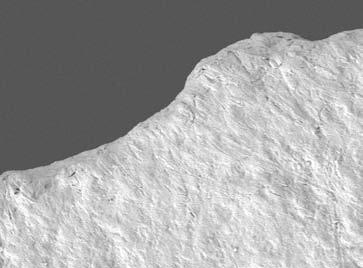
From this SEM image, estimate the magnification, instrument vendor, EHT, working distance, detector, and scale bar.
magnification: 1.5 K X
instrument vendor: JEOL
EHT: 5 kV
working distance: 9.6 mm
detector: LEI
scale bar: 10000 nm